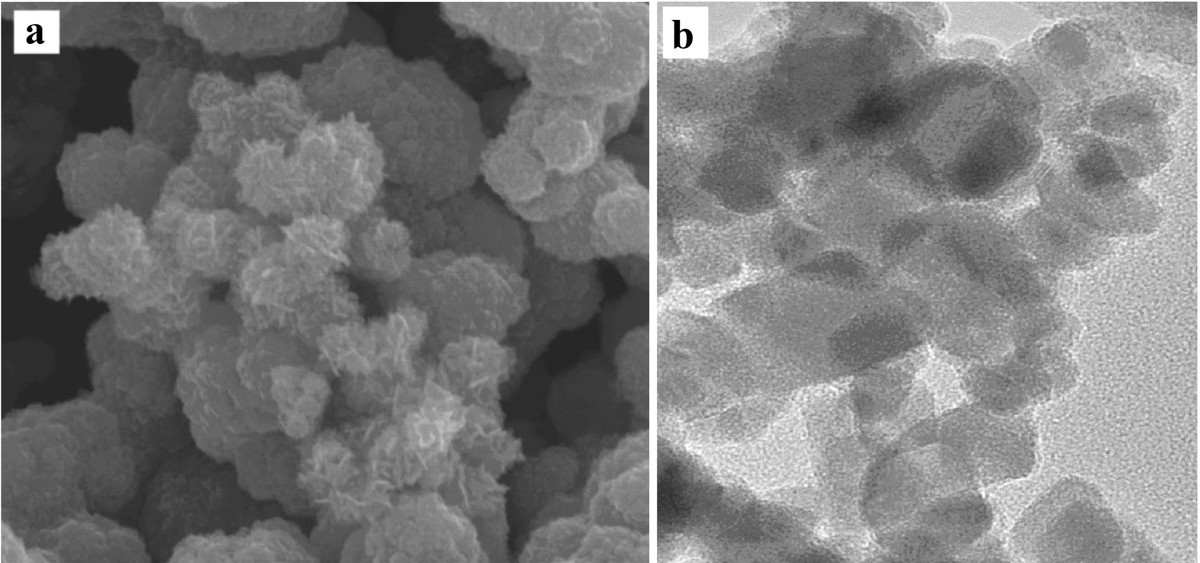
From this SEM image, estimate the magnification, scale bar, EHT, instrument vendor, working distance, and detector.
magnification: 100 K X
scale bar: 500 nm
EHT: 10 kV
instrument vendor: FEI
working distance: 5.7 mm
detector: TLD